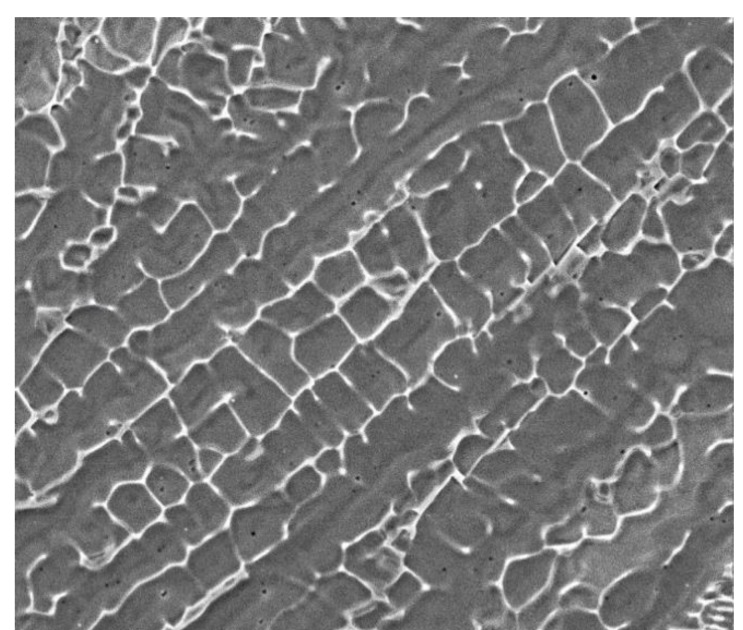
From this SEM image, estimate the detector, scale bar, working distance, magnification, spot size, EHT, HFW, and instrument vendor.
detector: SE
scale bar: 20000 nm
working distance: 10.5 mm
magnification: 5 K X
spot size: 4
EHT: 20 kV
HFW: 60 µm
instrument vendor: FEI